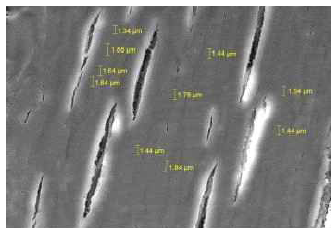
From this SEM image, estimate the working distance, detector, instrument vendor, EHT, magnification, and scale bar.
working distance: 10.23 mm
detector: SE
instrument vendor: Tescan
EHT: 10 kV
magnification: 5 K X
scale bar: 10000 nm